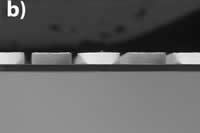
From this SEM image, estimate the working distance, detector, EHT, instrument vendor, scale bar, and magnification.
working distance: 4.9 mm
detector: BSE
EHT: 10 kV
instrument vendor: Hitachi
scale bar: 100000 nm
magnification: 0.3 K X